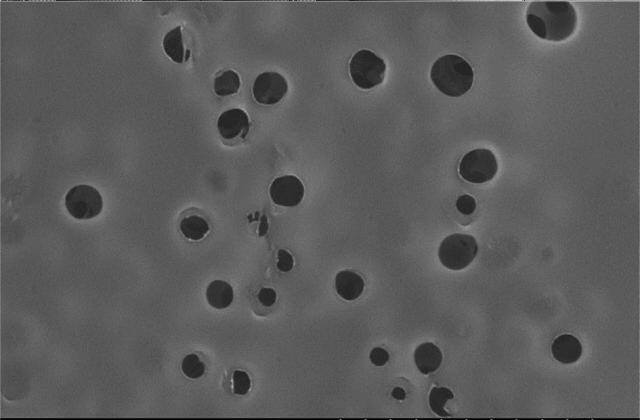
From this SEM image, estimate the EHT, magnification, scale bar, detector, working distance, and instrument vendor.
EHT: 5 kV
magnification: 10 K X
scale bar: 5000 nm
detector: SE(M,LA0)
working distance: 14.4 mm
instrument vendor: Hitachi